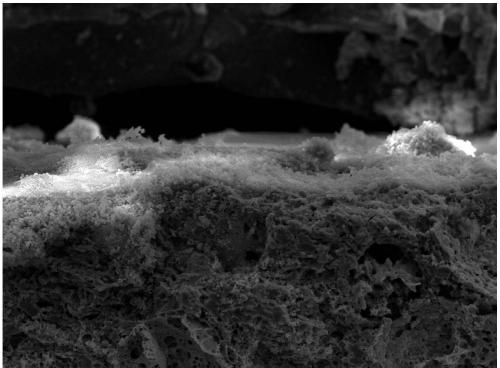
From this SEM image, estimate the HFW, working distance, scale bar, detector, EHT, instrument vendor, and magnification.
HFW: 277 µm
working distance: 12.24 mm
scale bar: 50000 nm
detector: SE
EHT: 15 kV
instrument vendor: Tescan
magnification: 1 K X